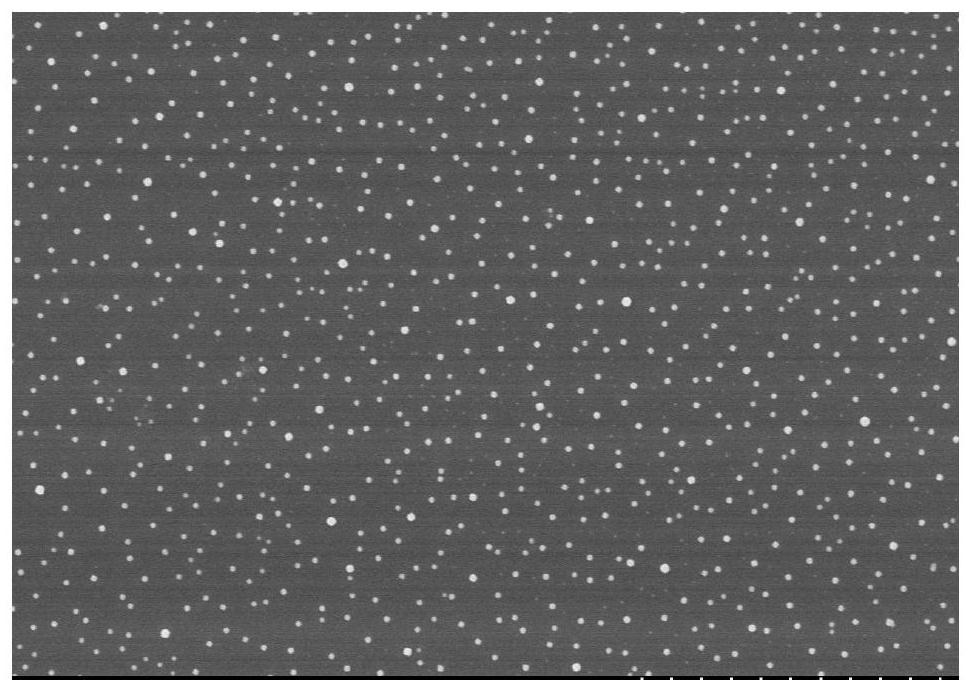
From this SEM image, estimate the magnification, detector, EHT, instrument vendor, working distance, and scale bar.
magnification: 20 K X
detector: SE(U,LA0)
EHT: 3 kV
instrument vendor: Hitachi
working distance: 8.3 mm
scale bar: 2000 nm